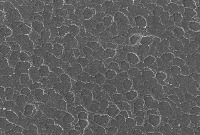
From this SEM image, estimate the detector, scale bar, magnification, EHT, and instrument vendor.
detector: SEI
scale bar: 100000 nm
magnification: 0.18 K X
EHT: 30 kV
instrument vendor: JEOL